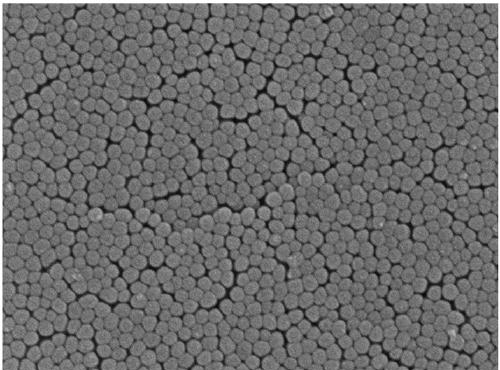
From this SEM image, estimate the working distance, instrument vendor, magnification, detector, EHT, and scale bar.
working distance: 6.6 mm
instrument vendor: JEOL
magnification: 100 K X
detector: SEI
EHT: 5 kV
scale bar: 100 nm